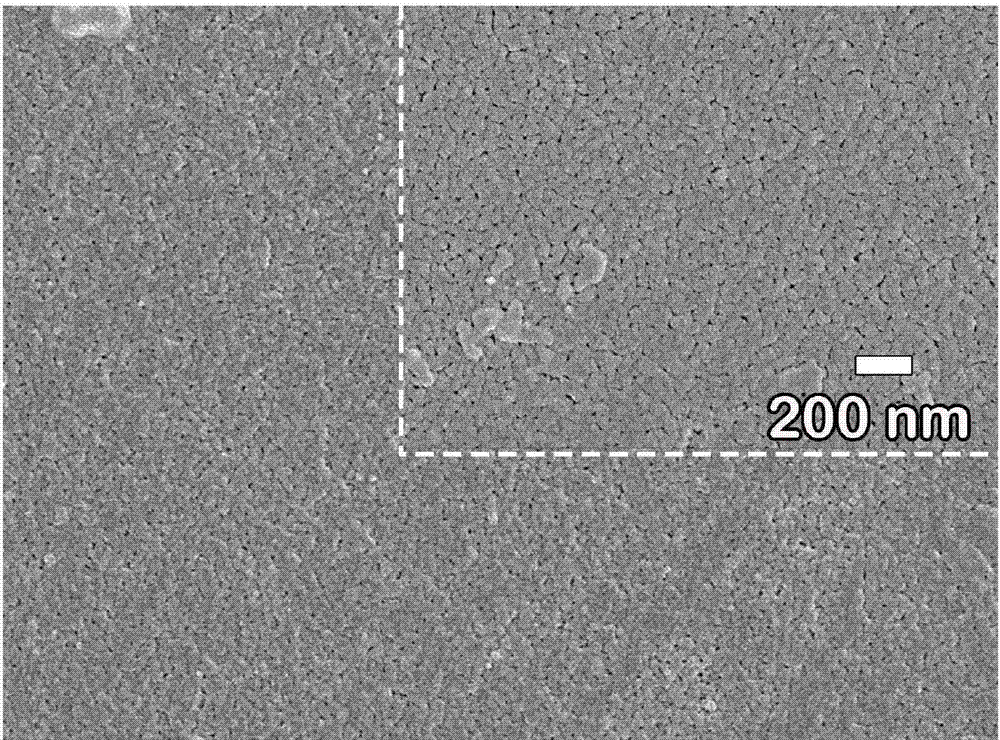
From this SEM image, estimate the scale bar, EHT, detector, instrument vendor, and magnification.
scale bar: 1000 nm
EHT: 5 kV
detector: SEI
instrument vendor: JEOL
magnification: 20 K X